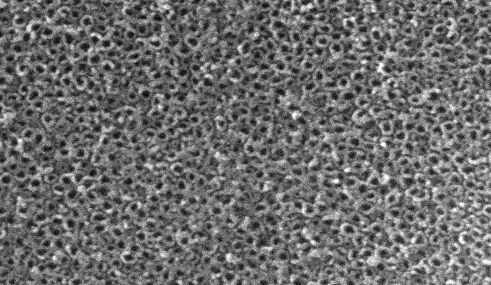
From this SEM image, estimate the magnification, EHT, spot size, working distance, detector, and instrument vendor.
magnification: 20 K X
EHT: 20 kV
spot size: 2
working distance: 5.1 mm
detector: SE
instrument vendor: FEI